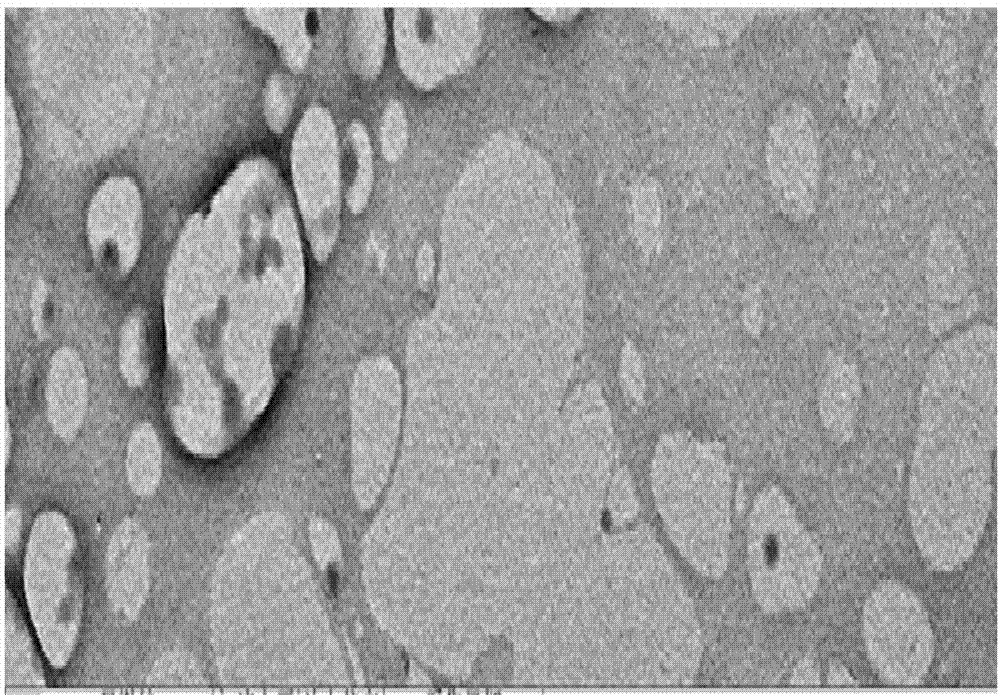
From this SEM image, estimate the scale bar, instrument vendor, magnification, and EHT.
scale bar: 500 nm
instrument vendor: Hitachi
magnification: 30 K X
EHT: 75 kV